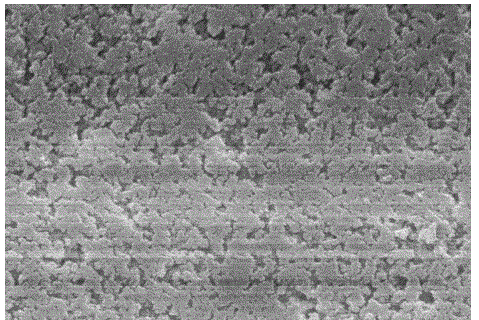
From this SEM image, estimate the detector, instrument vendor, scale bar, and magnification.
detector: SEI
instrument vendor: JEOL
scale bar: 1000 nm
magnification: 15 K X